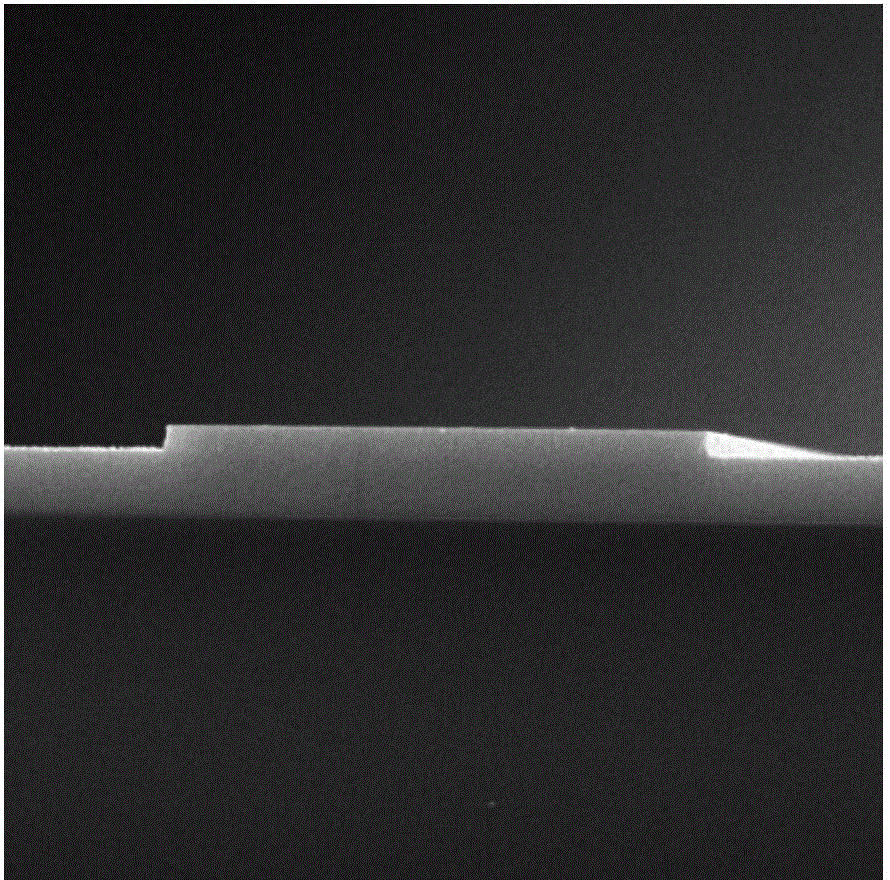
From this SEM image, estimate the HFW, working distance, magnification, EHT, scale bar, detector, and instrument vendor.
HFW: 12.9 µm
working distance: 7.74 mm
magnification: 23.4 K X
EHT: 15 kV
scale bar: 2000 nm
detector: SE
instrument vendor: Tescan